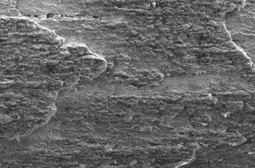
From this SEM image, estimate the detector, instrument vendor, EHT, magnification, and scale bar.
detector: SEI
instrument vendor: JEOL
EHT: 30 kV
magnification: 0.1 K X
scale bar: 100000 nm